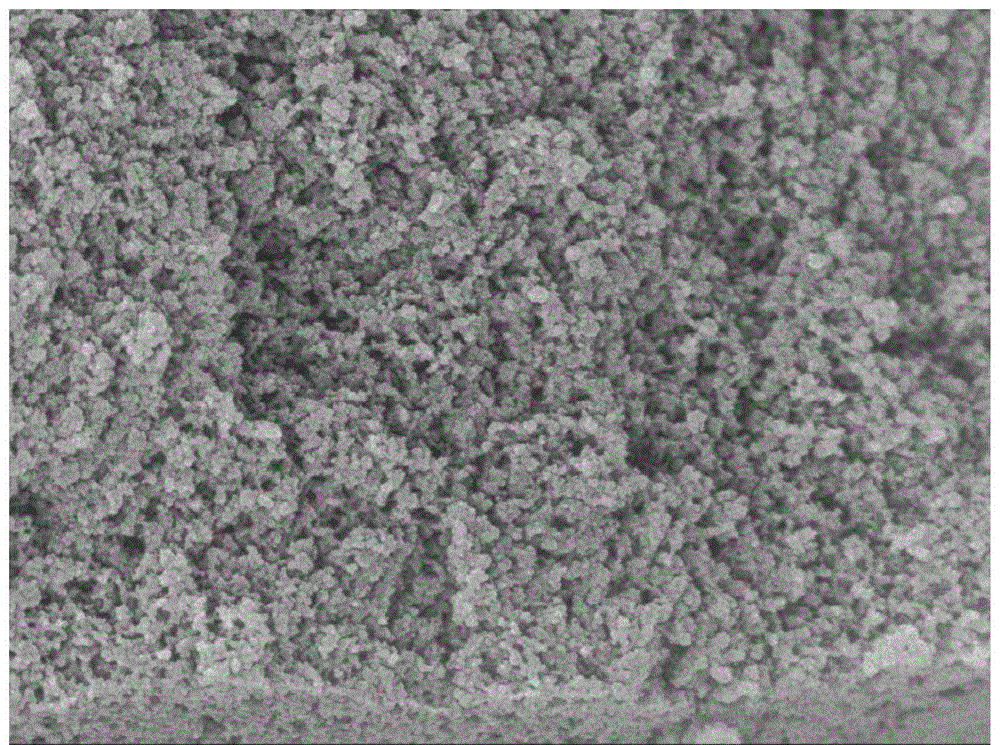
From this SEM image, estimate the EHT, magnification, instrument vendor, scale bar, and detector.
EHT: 5 kV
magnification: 50 K X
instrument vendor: JEOL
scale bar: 100 nm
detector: SEI